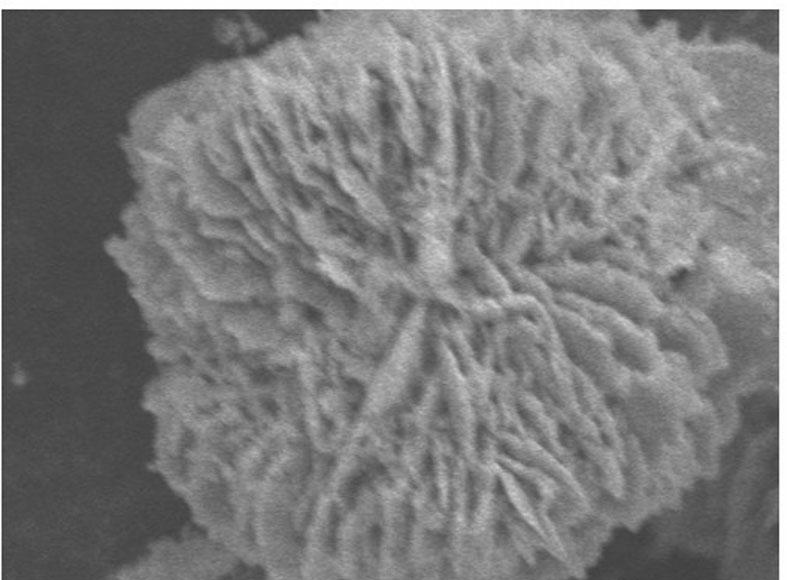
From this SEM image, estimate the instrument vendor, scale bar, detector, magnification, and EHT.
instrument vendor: JEOL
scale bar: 5000 nm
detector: SEI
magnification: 2.7 K X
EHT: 15 kV